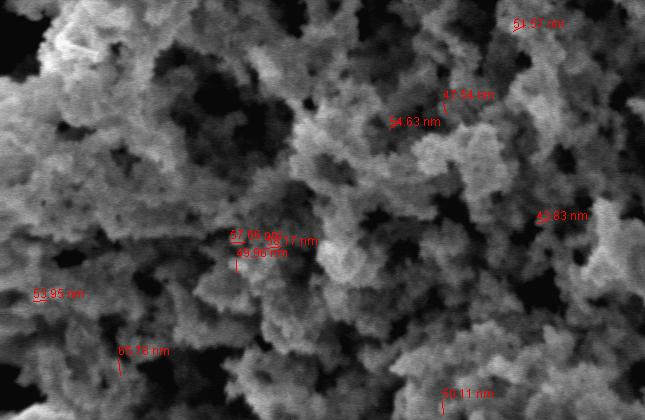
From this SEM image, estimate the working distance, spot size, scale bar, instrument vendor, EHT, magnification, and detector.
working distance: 5.6 mm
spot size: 2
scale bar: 500 nm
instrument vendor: FEI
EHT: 2 kV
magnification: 50 K X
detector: TLD